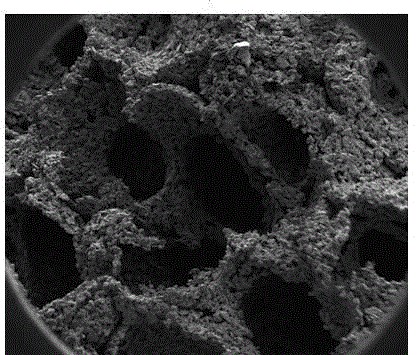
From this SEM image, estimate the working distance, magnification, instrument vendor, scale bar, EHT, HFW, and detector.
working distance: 8.1 mm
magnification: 0.1 K X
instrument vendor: FEI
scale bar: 500000 nm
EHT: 5 kV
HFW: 2400 µm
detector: ETD